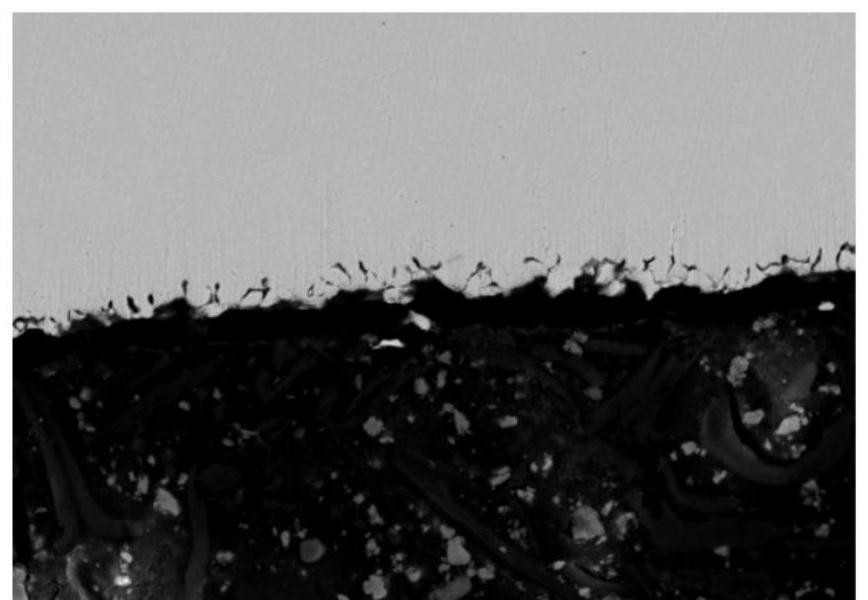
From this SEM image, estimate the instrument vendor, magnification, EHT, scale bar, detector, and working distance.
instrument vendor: Hitachi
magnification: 1 K X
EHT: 15 kV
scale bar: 50000 nm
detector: BSECOMP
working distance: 10.6 mm